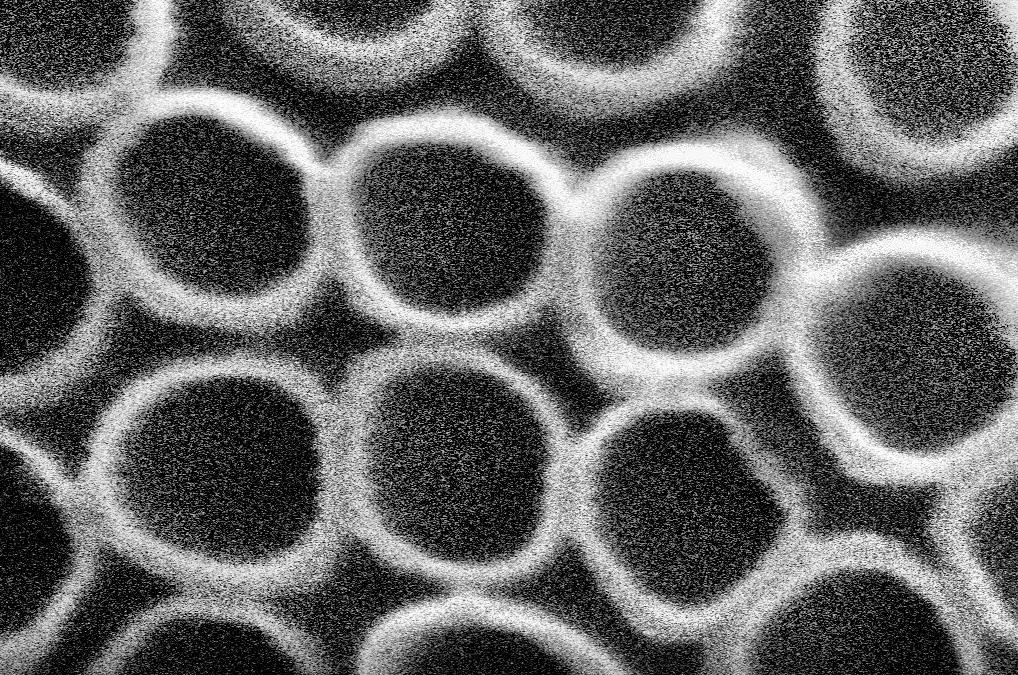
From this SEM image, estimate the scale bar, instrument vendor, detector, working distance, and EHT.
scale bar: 20 nm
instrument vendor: Zeiss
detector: InLens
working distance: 2.3 mm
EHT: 20 kV